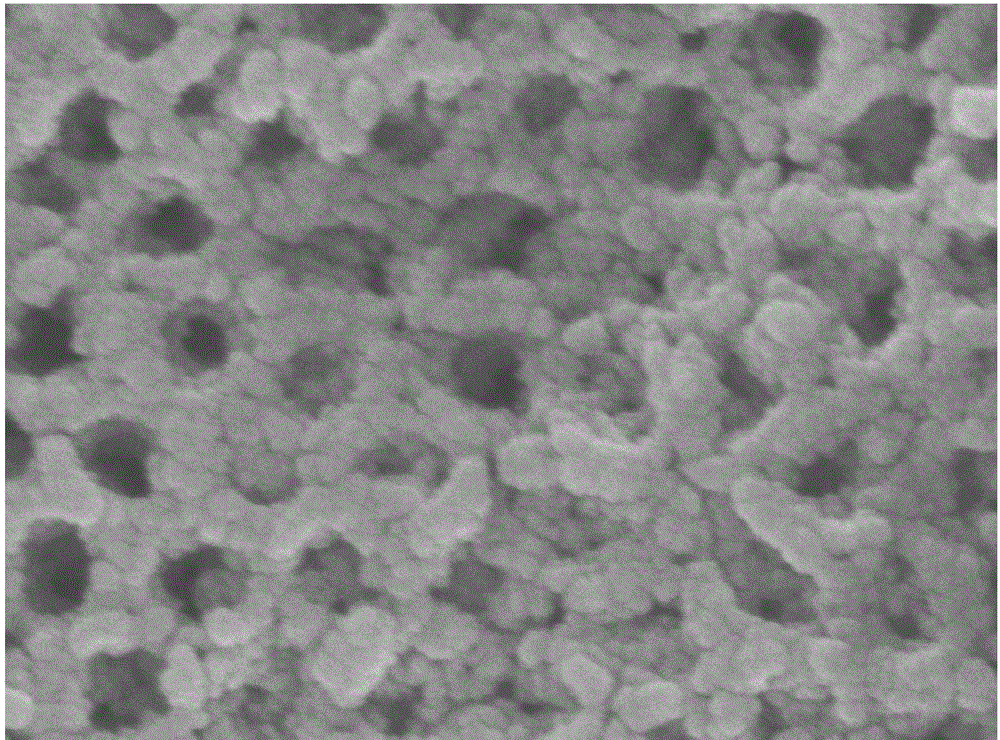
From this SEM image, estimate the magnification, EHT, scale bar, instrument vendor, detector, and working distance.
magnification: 50 K X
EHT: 8 kV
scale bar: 100 nm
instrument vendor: JEOL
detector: SEI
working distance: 7.9 mm